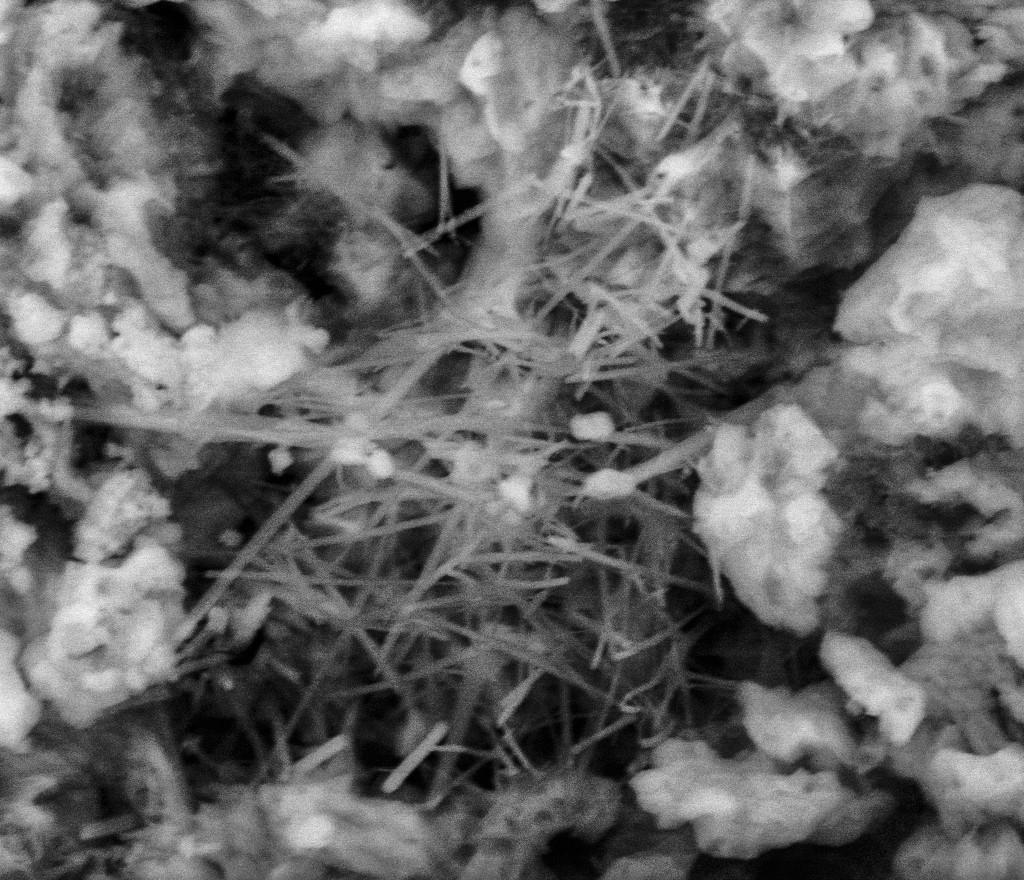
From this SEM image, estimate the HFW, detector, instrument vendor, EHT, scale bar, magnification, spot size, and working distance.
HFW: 9.95 µm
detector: BSED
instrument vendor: FEI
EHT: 10 kV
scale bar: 3000 nm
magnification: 15 K X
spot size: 5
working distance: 4.3 mm